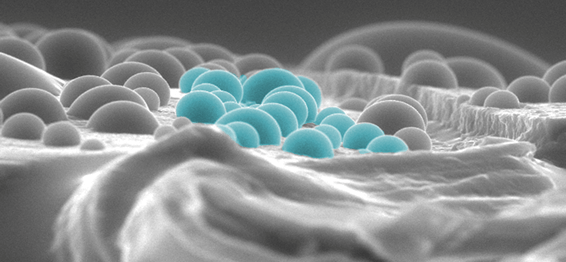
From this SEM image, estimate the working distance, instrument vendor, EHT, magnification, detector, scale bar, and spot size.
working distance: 10.1 mm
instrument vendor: FEI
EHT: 30 kV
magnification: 0.5 K X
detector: GSE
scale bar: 50000 nm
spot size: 5.2